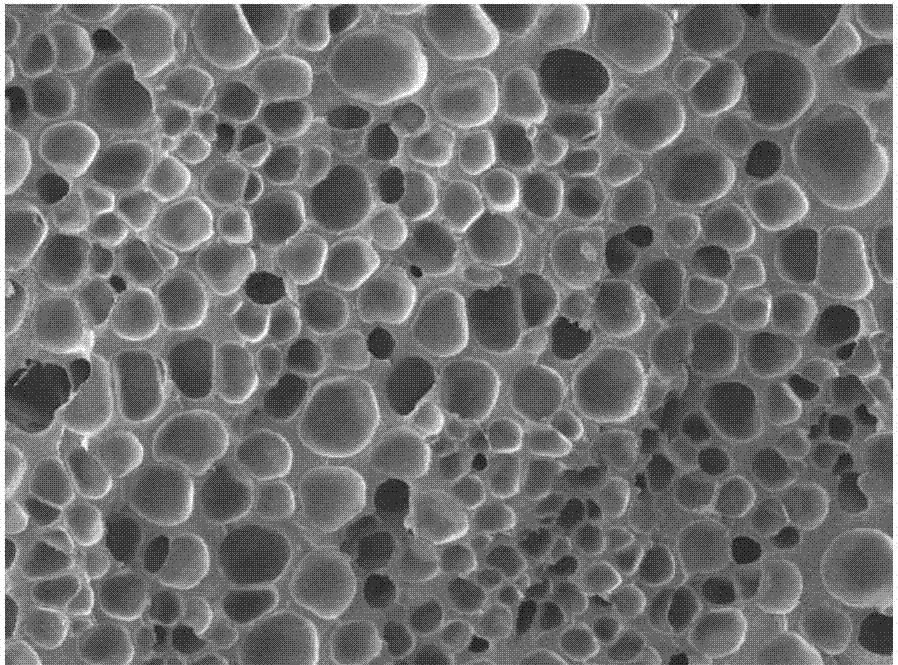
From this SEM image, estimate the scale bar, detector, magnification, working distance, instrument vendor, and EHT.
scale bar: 100000 nm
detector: SEI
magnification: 0.05 K X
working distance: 8 mm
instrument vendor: JEOL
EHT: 5 kV